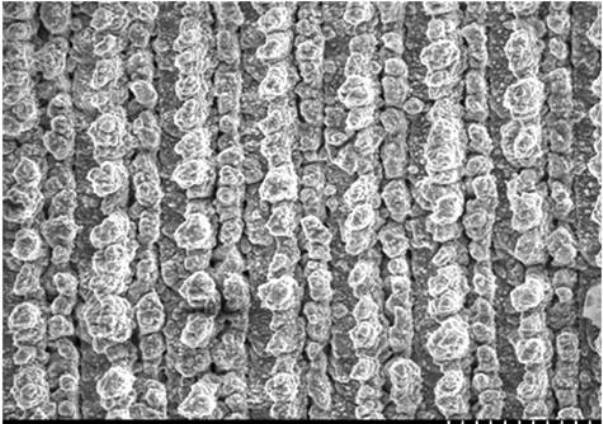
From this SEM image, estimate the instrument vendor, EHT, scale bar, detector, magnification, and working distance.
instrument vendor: Hitachi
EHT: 10 kV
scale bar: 100000 nm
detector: SE(M)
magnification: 0.3 K X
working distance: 10.7 mm